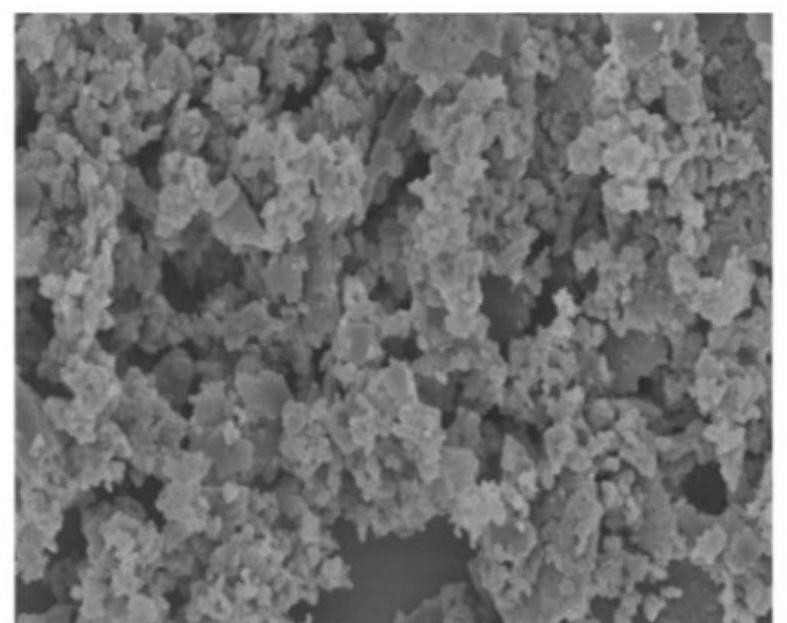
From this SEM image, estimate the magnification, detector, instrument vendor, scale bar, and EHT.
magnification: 5 K X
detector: SE(M)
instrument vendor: Hitachi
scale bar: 10000 nm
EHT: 5 kV